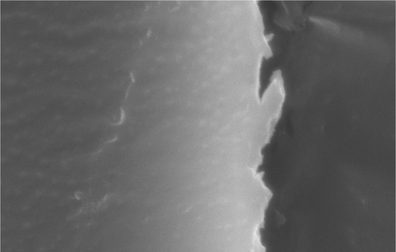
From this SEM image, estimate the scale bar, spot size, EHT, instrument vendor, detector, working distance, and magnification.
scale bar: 2000 nm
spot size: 3.8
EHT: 20 kV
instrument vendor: FEI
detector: SE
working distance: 11.6 mm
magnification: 10 K X